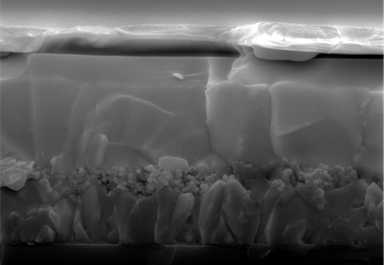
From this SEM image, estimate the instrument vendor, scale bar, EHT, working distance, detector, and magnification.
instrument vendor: Hitachi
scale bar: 1000 nm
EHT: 5 kV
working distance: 8.6 mm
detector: SE(U)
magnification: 30 K X